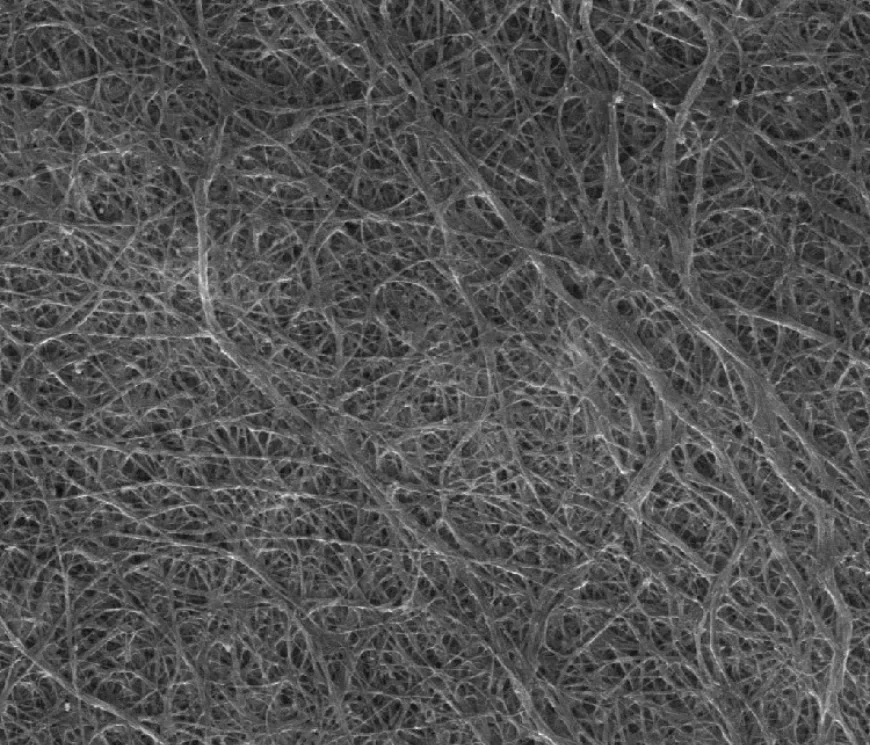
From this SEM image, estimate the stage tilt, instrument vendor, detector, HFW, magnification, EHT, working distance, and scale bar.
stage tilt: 0°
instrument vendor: FEI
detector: ETD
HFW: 9.95 µm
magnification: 30 K X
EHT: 10 kV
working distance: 11.7 mm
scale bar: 2000 nm